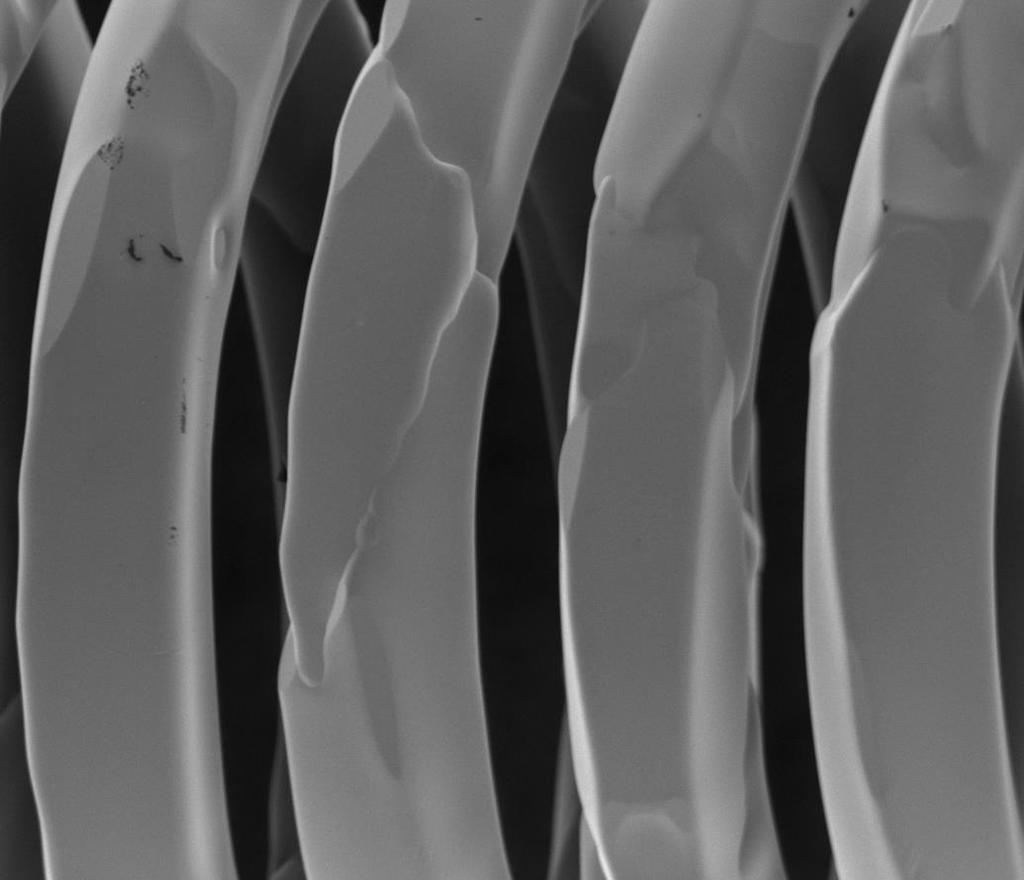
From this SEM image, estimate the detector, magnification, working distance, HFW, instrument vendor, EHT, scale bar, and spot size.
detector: ETD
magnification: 2.004 K X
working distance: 3.2 mm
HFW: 149 µm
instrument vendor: FEI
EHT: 1 kV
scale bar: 50000 nm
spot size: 5